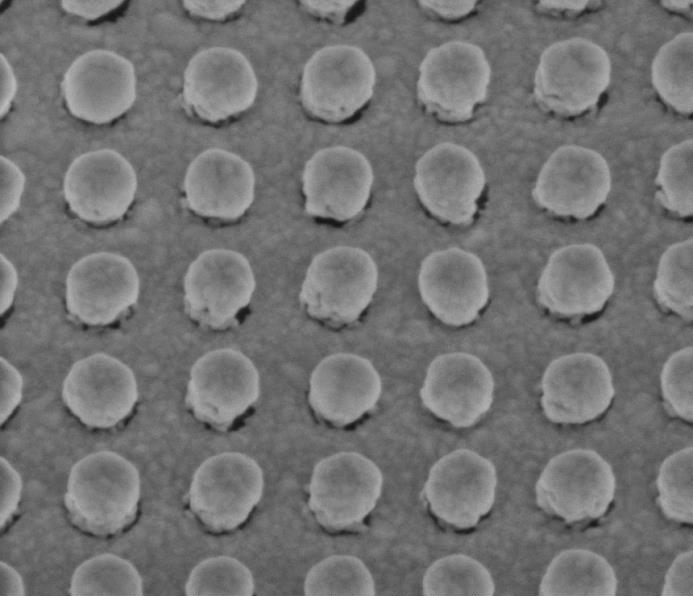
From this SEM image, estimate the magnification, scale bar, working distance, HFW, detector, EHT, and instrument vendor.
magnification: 100 K X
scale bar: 200 nm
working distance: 5 mm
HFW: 0.995 µm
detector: TLD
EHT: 2 kV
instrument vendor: FEI